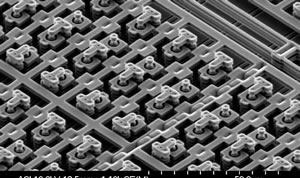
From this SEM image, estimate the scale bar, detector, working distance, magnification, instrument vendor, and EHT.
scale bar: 50000 nm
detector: SE(M)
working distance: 12.5 mm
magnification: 1.1 K X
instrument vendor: Hitachi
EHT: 10 kV